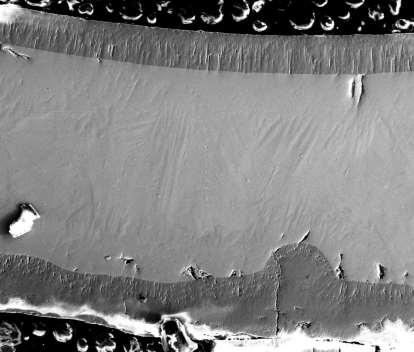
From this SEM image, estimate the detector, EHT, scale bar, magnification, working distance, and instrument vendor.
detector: ETD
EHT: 20 kV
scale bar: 400000 nm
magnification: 0.15 K X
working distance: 10.1 mm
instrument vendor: FEI